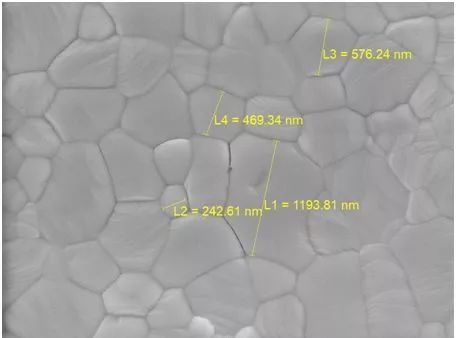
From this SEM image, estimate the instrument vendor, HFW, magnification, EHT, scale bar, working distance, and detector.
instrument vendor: Tescan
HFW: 4.61 µm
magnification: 60 K X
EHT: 5 kV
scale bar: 1000 nm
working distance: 5 mm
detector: In-Beam SE